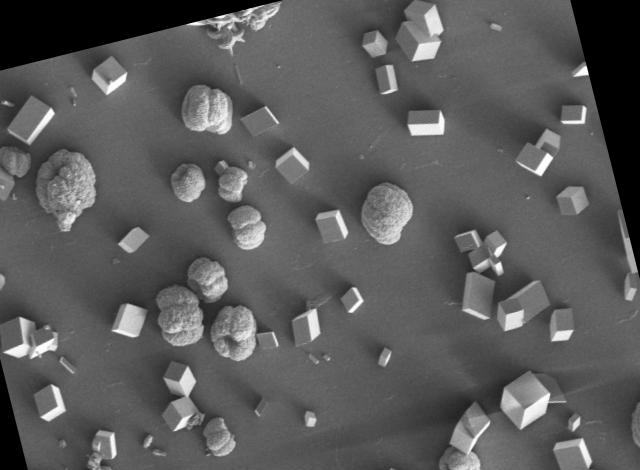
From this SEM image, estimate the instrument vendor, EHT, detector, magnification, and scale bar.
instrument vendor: Tescan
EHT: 5 kV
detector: SE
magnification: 0.4 K X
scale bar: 200000 nm